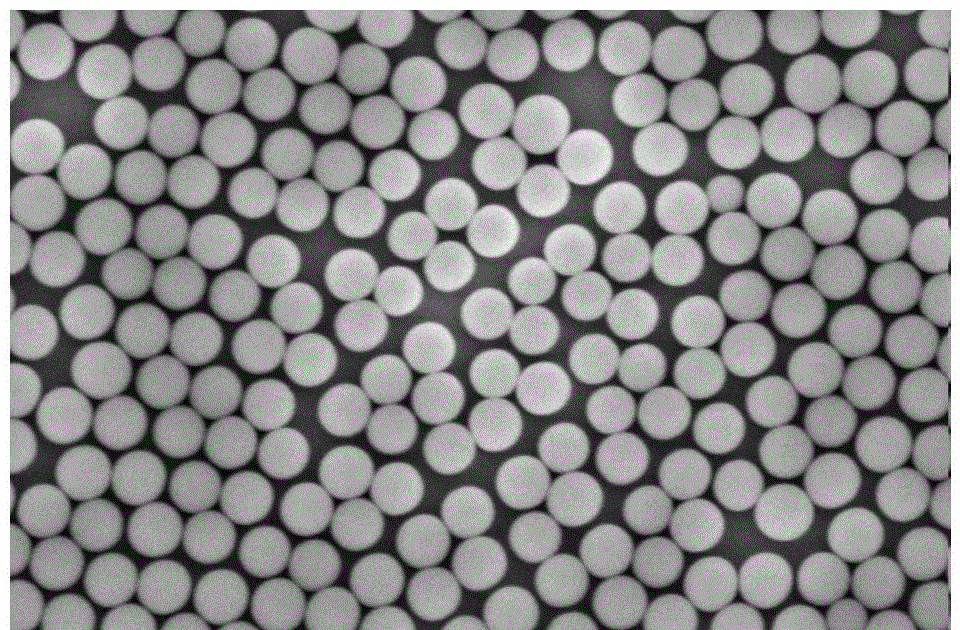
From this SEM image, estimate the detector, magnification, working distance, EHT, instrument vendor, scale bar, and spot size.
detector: TLD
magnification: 50 K X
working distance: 3.1 mm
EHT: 5 kV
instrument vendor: FEI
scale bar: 1000 nm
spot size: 4